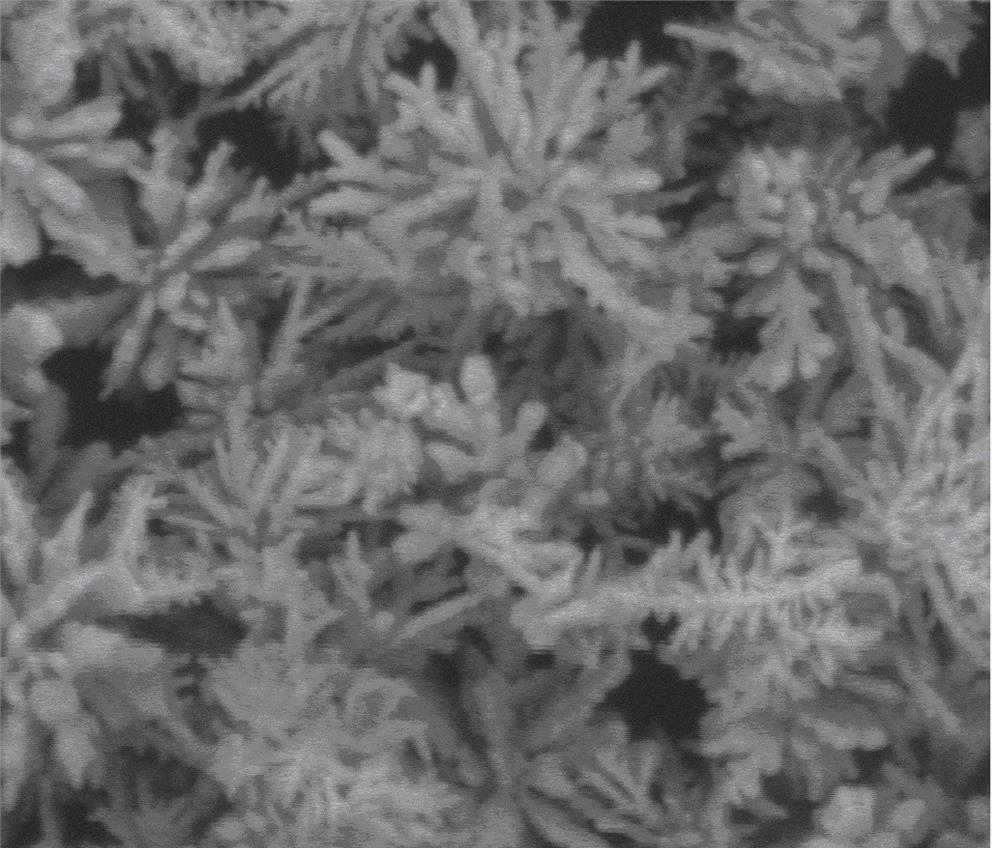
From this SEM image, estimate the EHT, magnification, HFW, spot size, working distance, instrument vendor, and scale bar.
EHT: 20 kV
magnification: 5 K X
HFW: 29.8 µm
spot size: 3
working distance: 9.9 mm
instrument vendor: FEI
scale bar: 10000 nm